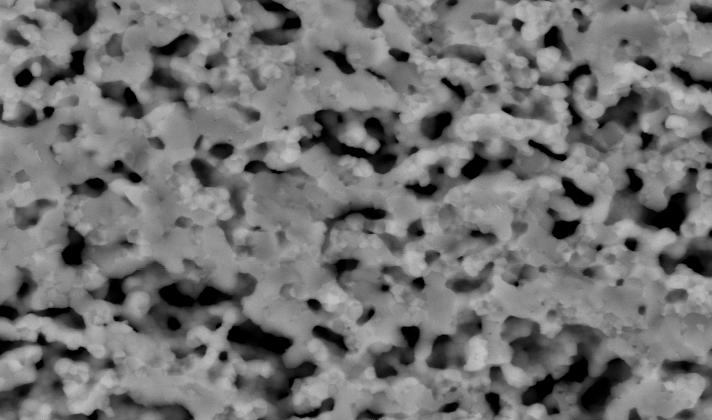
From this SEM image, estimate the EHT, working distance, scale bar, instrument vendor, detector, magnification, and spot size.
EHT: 20 kV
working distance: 7.4 mm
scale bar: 5000 nm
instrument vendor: FEI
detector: BSE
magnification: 5 K X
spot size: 3